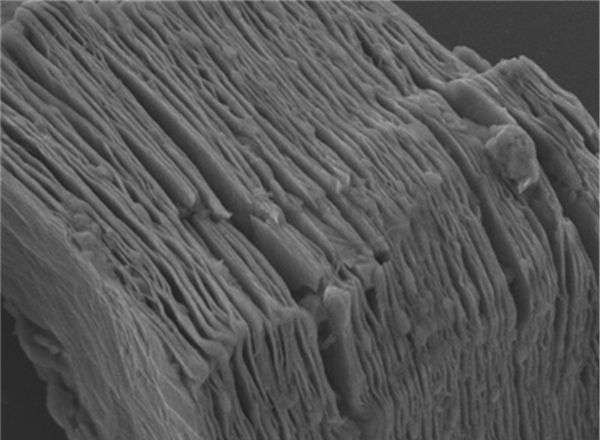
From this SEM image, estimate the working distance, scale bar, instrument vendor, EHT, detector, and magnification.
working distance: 10.3 mm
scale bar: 1000 nm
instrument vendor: JEOL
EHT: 4 kV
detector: LED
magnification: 20 K X